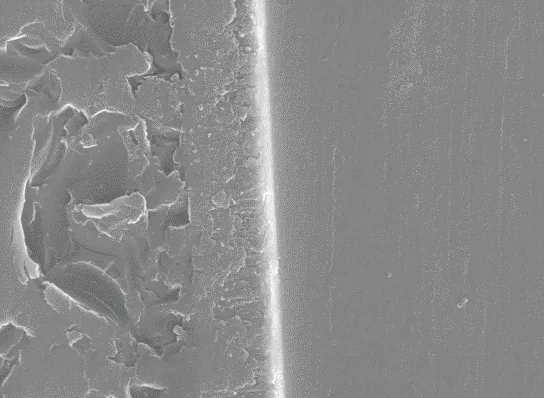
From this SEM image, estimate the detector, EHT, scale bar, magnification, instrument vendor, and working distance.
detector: SE1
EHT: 25 kV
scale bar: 1000 nm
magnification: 10 K X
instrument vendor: Zeiss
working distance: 7.5 mm